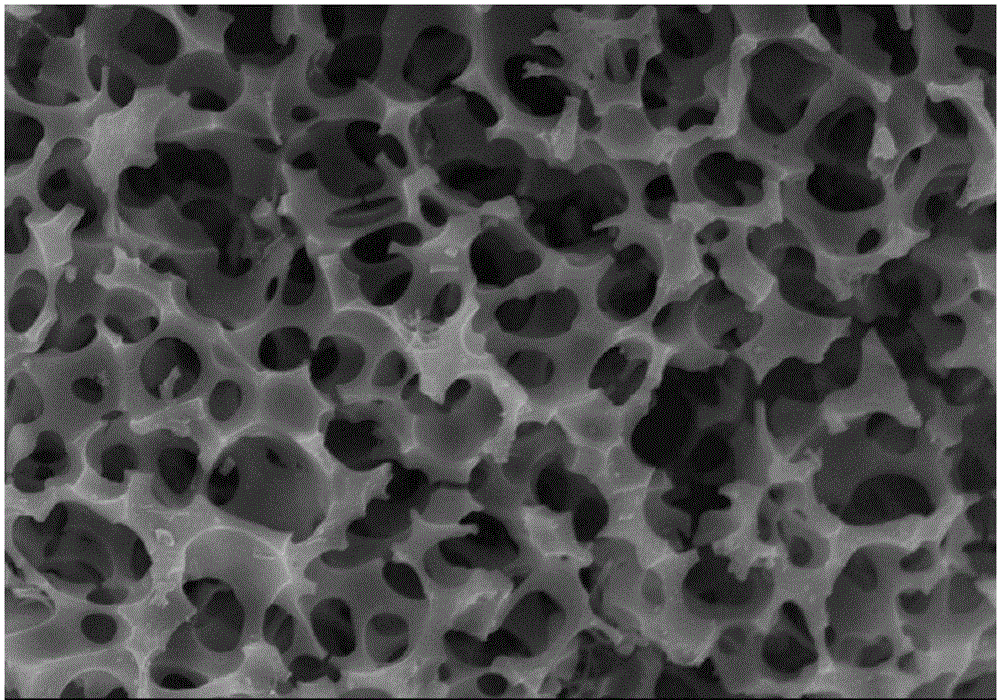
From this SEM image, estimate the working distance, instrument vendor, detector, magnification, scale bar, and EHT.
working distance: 14.8 mm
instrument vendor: Hitachi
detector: SE(M,LA0)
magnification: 5 K X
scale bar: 10000 nm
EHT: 10 kV